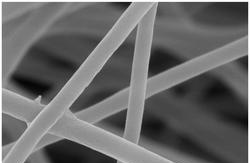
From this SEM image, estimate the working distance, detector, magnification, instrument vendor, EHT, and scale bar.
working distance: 9.3 mm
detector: InLens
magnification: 50 K X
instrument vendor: Zeiss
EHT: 5 kV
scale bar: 200 nm